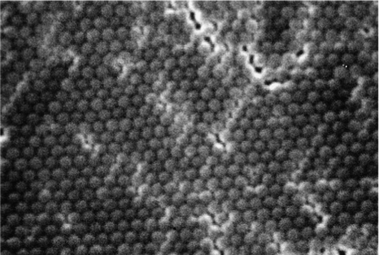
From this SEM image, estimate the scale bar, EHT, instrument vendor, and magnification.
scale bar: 200 nm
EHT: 20 kV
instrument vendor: JEOL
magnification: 50 K X